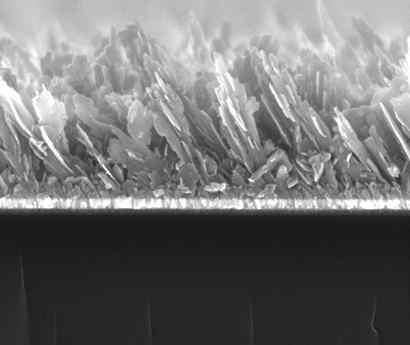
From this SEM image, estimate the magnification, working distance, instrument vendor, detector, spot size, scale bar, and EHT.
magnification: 100 K X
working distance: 7.8 mm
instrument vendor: FEI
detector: ETD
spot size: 3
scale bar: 500 nm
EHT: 10 kV